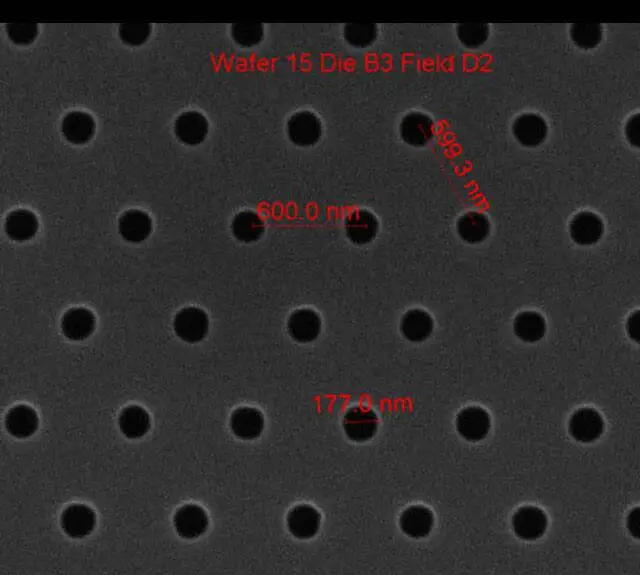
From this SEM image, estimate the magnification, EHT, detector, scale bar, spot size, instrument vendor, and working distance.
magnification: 75 K X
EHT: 10 kV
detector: ETD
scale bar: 1000 nm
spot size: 1.5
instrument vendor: FEI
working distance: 10.3 mm